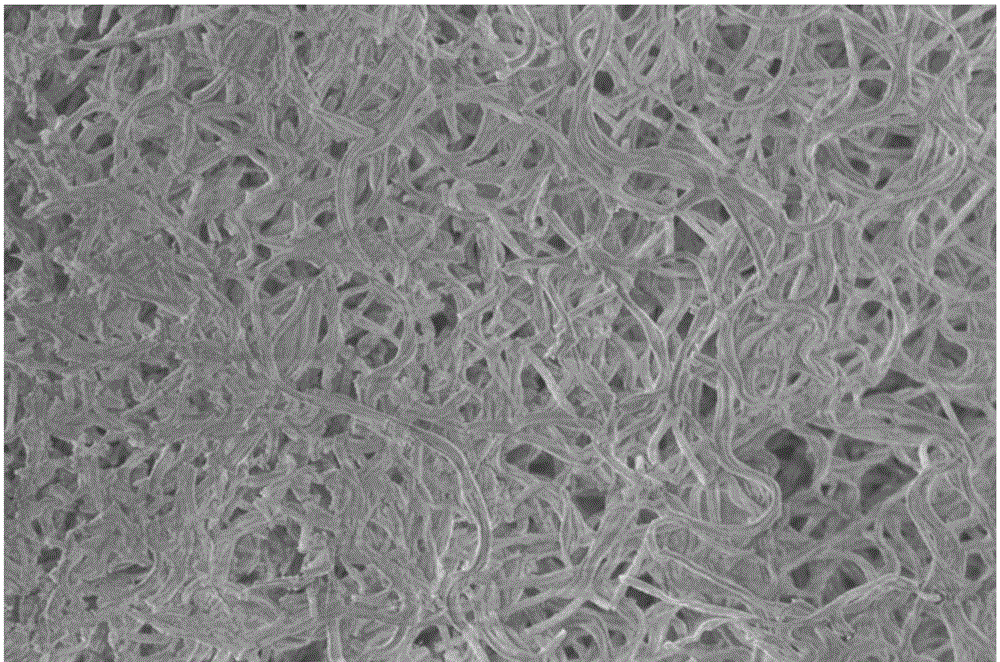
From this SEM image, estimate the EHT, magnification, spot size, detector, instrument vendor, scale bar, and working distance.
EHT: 2 kV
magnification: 5 K X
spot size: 2.5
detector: TLD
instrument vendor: FEI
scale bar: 10000 nm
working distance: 6.5 mm